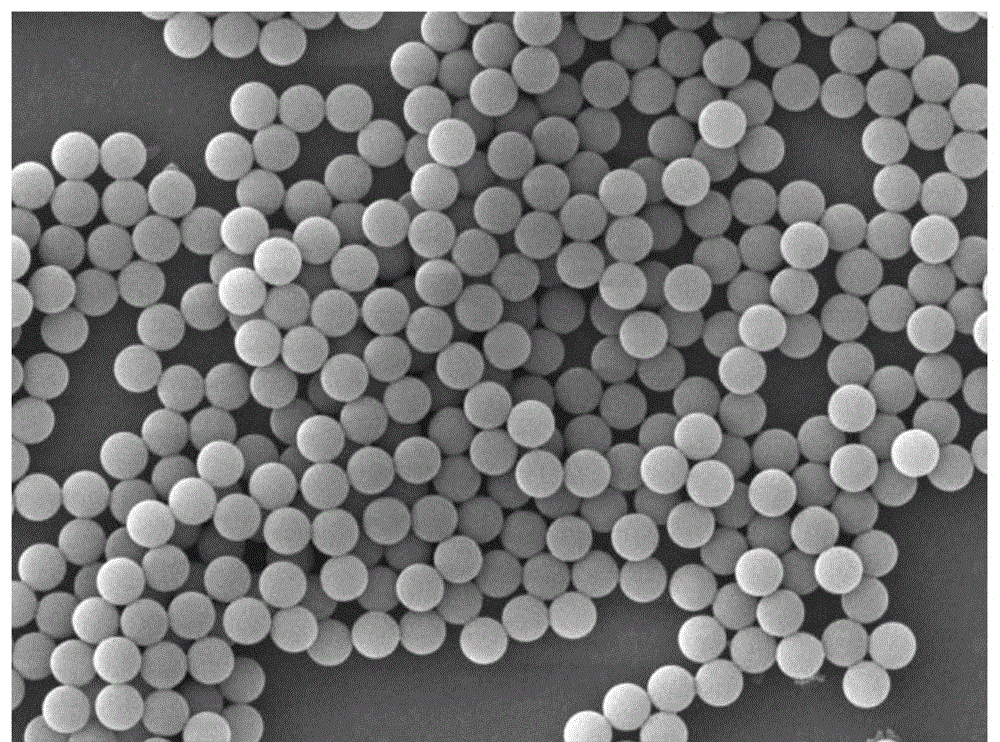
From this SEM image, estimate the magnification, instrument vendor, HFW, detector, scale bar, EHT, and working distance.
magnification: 5 K X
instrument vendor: Tescan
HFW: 55.4 µm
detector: SE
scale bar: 10000 nm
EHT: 20 kV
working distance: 8.97 mm